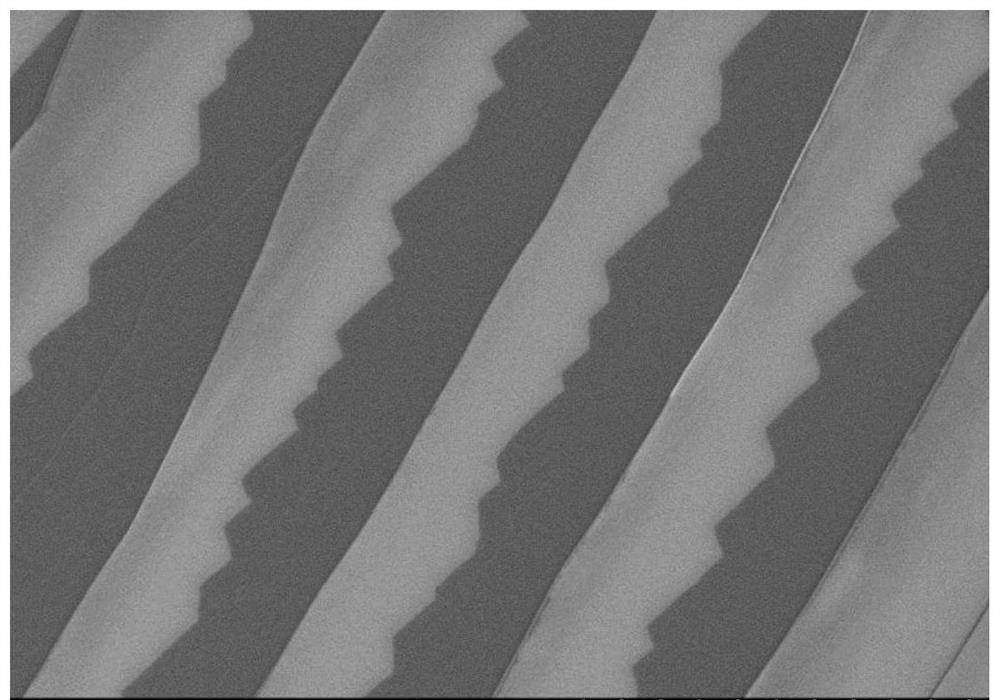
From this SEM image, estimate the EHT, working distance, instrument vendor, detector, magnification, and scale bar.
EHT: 5 kV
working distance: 10.1 mm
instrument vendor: Hitachi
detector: SE(M)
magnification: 5 K X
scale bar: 10000 nm